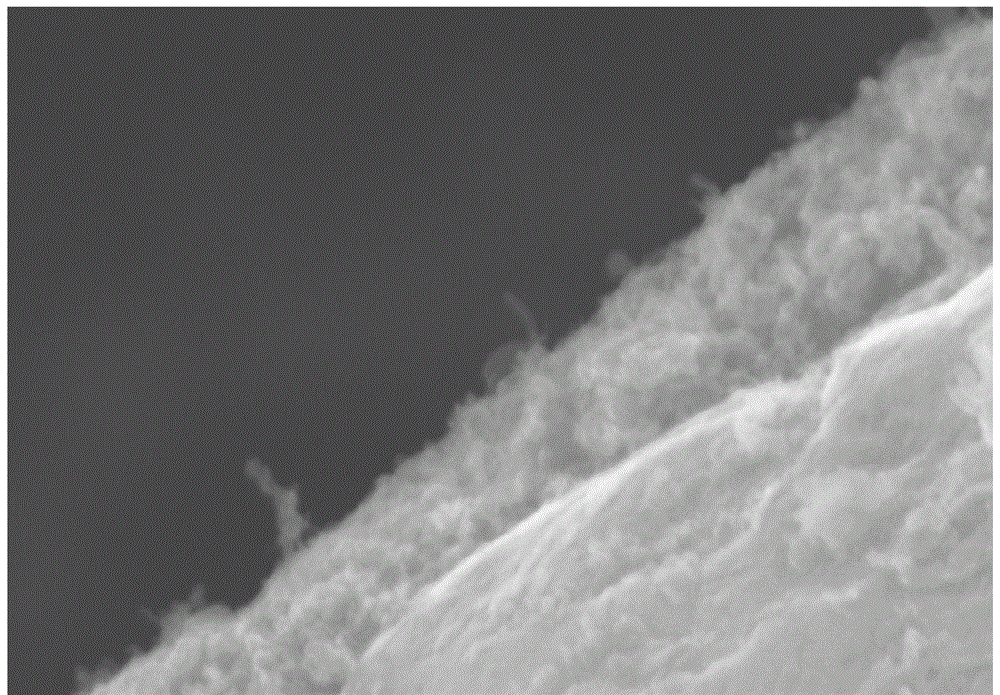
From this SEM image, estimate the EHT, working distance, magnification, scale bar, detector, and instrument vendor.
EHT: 15 kV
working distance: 18.6 mm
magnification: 50 K X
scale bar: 1000 nm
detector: SE(M)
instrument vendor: Hitachi